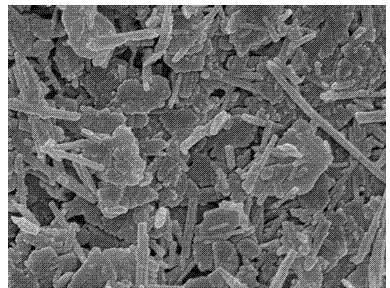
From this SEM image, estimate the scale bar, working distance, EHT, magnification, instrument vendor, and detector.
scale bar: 100 nm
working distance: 7.9 mm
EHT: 5 kV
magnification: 40 K X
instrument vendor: JEOL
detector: SEI